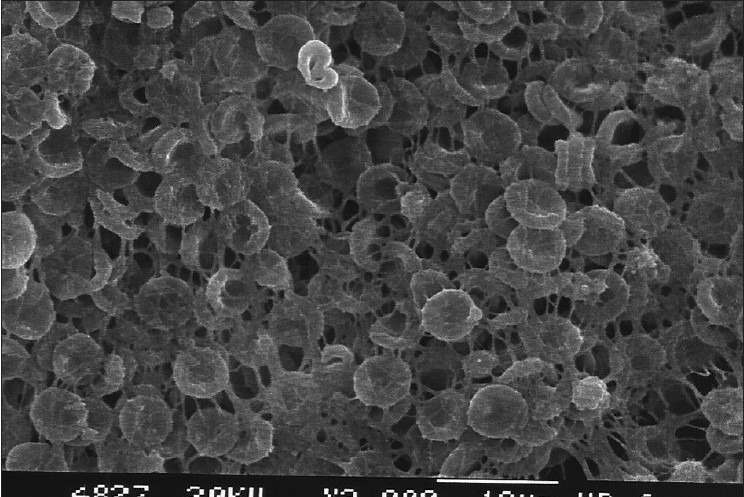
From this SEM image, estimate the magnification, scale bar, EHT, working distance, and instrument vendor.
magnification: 2 K X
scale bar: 10000 nm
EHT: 20 kV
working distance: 6 mm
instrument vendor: JEOL